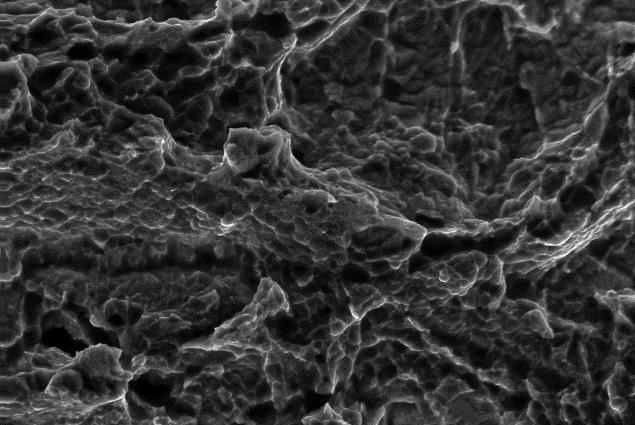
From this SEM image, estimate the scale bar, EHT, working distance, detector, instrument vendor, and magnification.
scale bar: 10000 nm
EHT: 20 kV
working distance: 23 mm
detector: SE1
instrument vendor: Zeiss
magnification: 2.5 K X